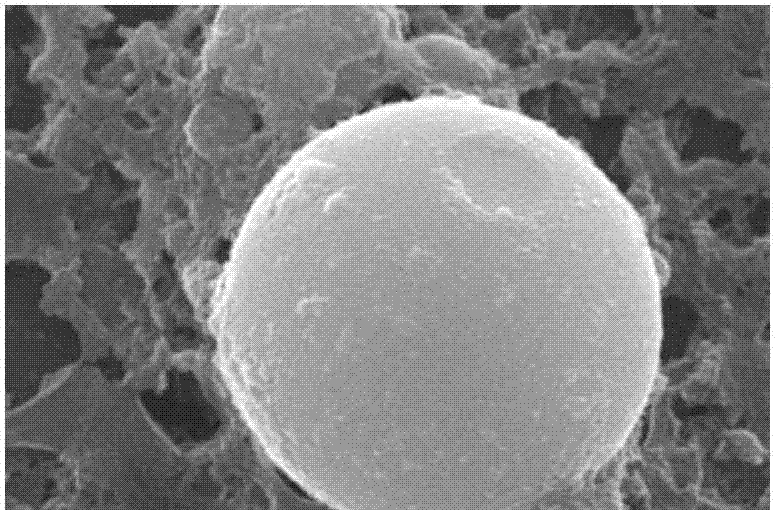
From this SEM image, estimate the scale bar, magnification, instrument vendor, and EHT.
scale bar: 1000 nm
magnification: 20 K X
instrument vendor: JEOL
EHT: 15 kV